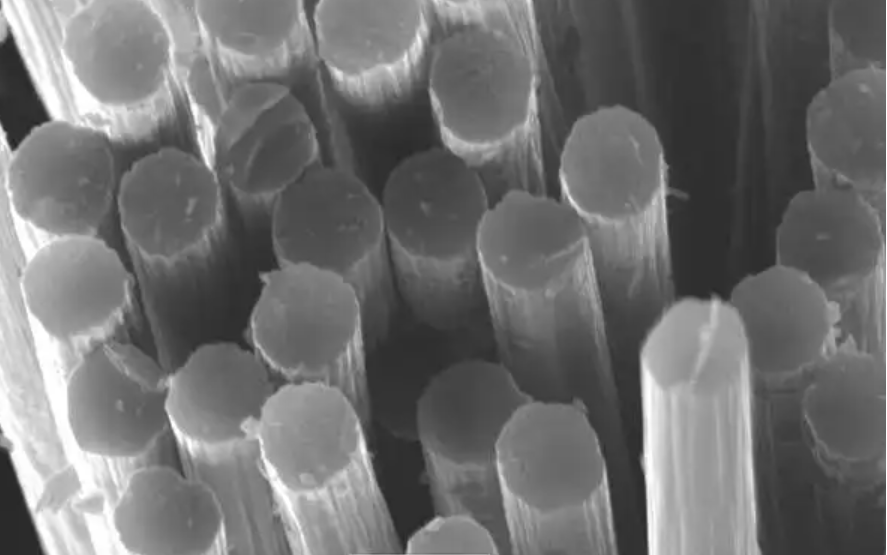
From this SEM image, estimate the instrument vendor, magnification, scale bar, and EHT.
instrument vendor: JEOL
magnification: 2 K X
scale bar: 10000 nm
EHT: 25 kV